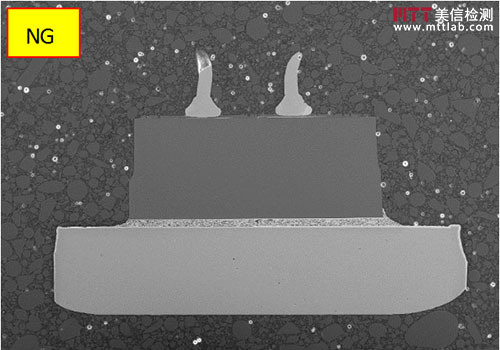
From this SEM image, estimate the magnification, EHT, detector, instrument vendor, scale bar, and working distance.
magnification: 0.15 K X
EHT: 15 kV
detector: SE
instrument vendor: Hitachi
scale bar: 300000 nm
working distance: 10 mm